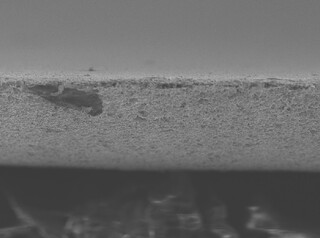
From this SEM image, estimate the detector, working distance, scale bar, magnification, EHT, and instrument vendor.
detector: SE(UL)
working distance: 9.9 mm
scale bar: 50000 nm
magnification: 1 K X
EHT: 10 kV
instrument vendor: Hitachi